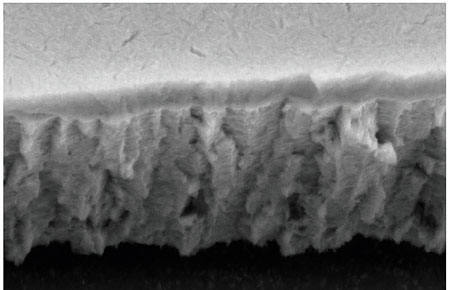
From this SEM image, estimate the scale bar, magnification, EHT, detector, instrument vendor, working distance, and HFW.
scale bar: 200 nm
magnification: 20 K X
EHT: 10 kV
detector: SE2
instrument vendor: Zeiss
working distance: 8.1 mm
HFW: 5.716 µm